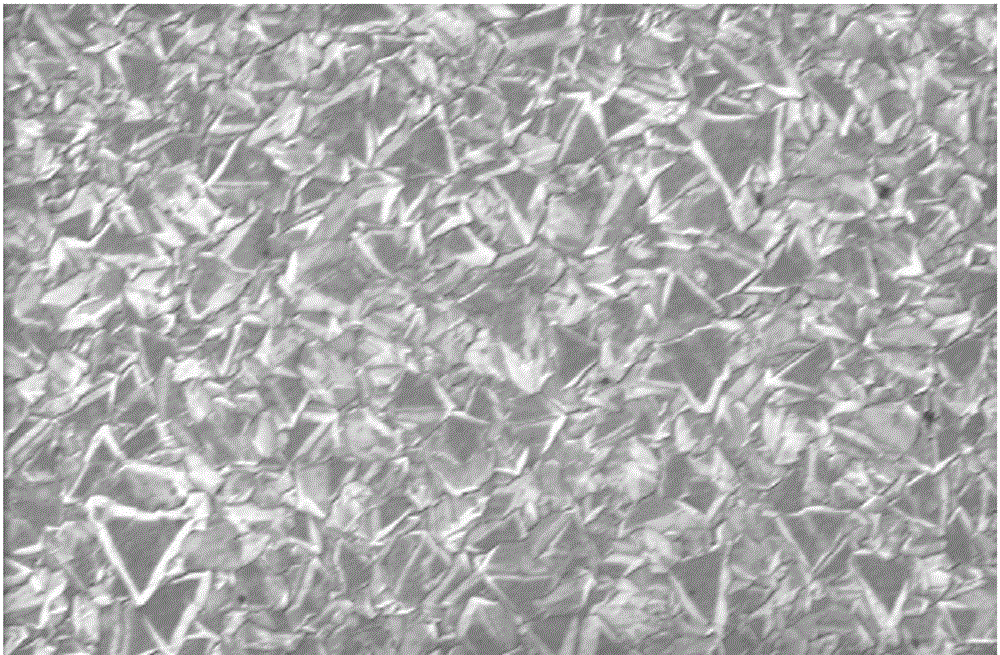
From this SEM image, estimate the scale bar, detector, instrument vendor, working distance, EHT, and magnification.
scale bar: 1000 nm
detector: InLens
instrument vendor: Zeiss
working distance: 5 mm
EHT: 3 kV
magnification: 15 K X